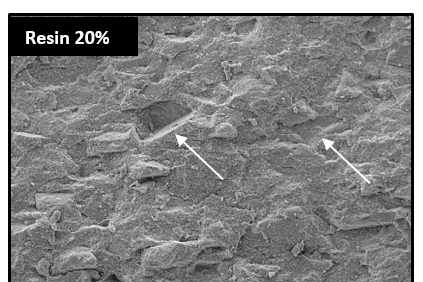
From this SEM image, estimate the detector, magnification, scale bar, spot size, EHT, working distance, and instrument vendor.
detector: BSE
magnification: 0.1 K X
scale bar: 200000 nm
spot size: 3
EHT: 15 kV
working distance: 10.4 mm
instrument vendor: FEI